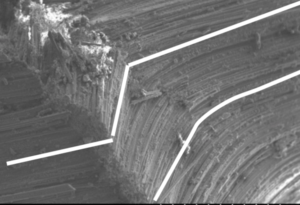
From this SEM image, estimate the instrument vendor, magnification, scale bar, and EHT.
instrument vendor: Hitachi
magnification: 0.05 K X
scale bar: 1000000 nm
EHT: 15 kV